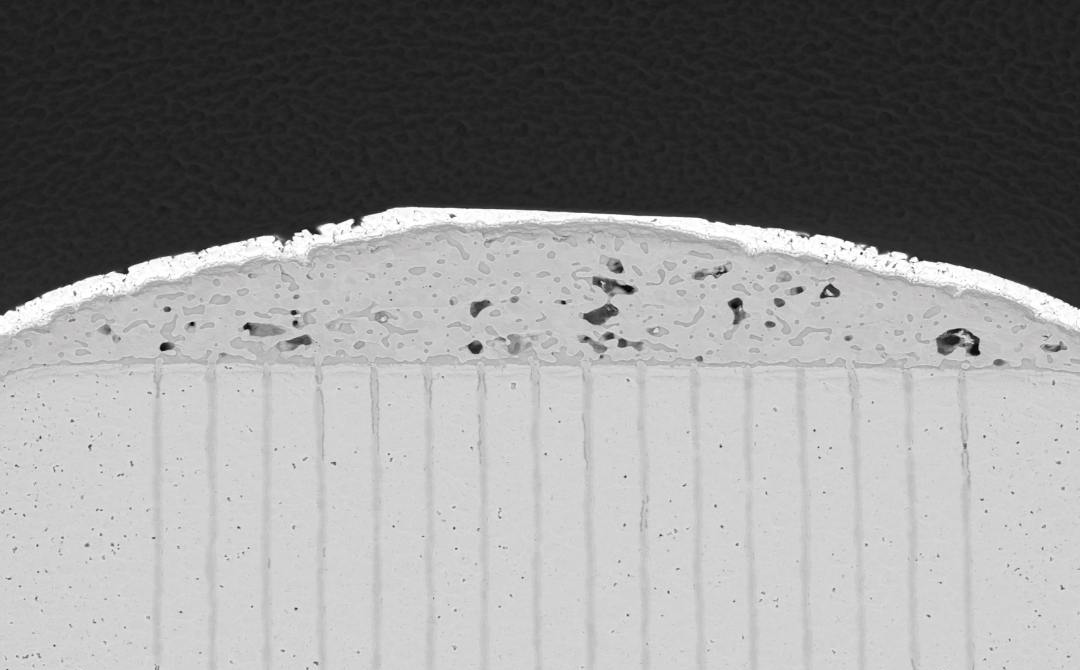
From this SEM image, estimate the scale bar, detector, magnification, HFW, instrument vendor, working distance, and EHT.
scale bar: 80000 nm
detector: BSD Full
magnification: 0.5 K X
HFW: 254 µm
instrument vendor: other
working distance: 9.168 mm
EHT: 15 kV